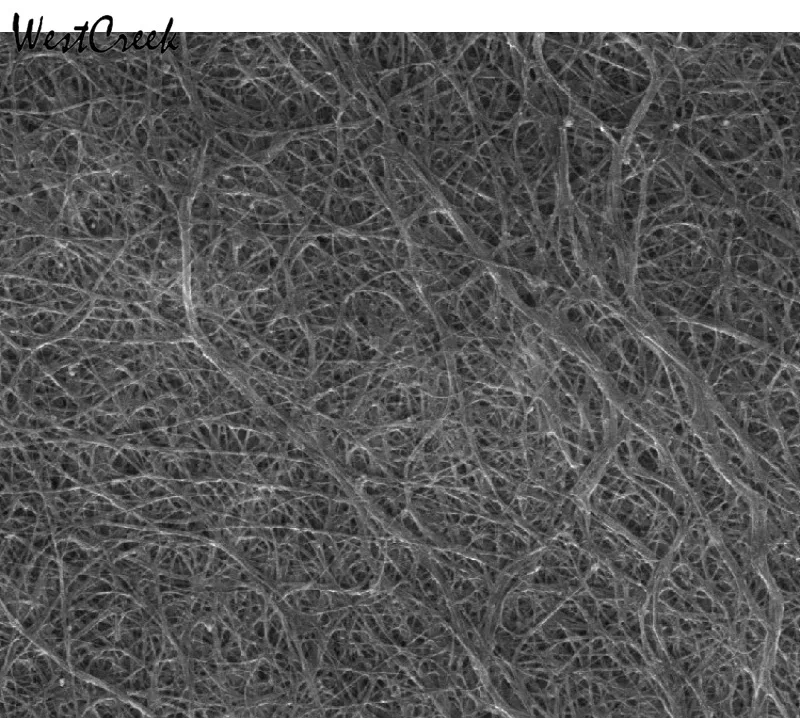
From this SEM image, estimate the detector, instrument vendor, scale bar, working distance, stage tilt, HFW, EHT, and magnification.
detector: ETD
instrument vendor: FEI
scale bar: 2000 nm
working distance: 11.7 mm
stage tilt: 0°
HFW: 9.95 µm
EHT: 10 kV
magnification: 30 K X